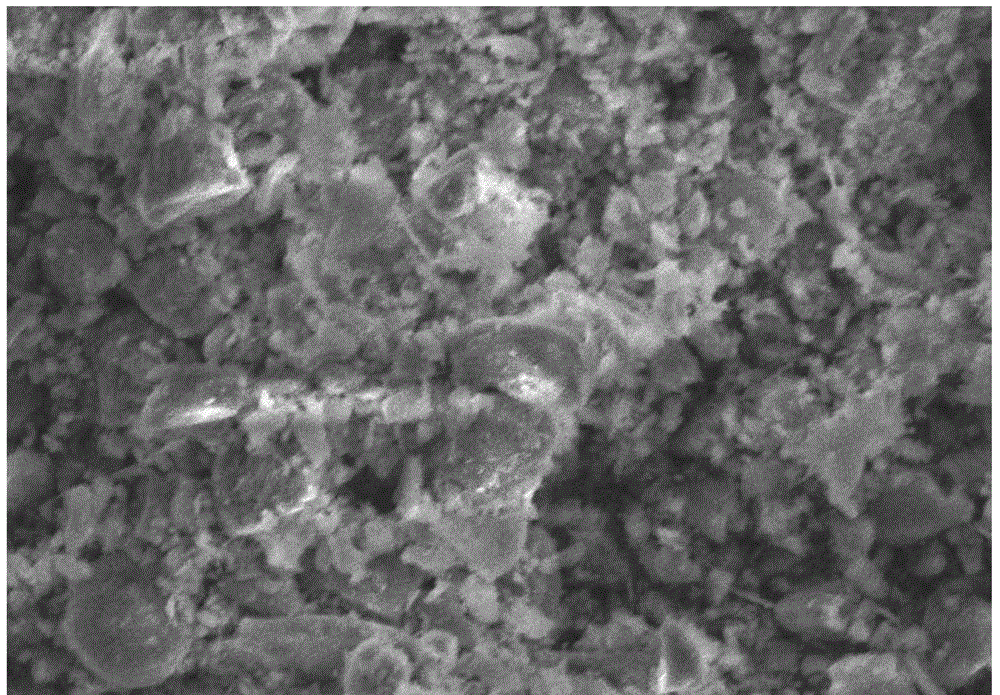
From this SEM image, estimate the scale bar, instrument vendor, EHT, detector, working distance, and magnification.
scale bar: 50000 nm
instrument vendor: Hitachi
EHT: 20 kV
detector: SE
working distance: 39.4 mm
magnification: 0.752 K X